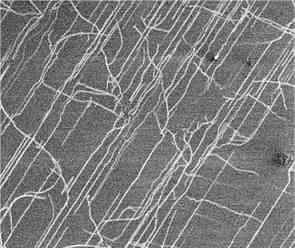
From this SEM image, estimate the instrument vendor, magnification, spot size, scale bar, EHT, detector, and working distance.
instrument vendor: FEI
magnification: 4.256 K X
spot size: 3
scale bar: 5000 nm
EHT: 2 kV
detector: SE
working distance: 4.7 mm